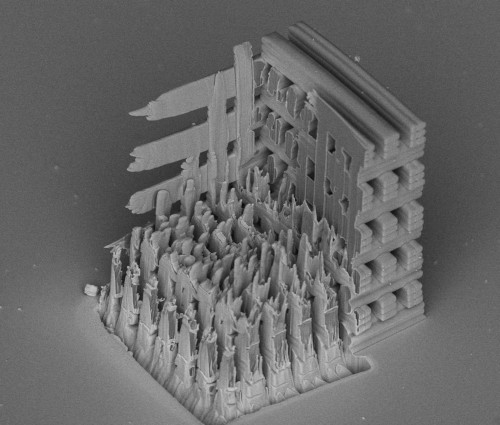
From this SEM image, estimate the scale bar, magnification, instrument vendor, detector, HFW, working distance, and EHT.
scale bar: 20000 nm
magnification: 2.275 K X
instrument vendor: FEI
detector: TLD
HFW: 121 µm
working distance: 4.1 mm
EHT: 5 kV